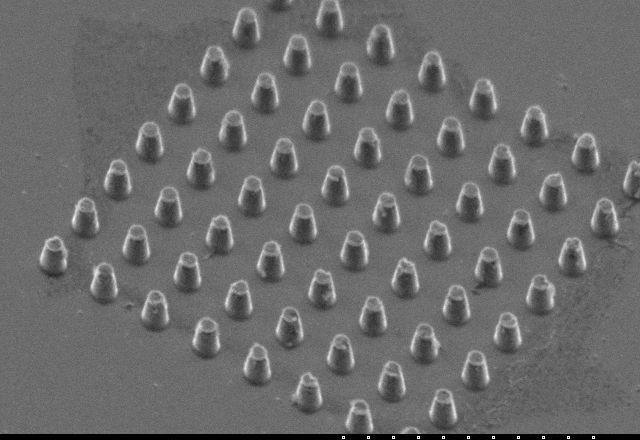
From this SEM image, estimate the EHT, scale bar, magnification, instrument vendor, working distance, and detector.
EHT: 5 kV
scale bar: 10000 nm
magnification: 5 K X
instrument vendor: Hitachi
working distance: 11.8 mm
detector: SE